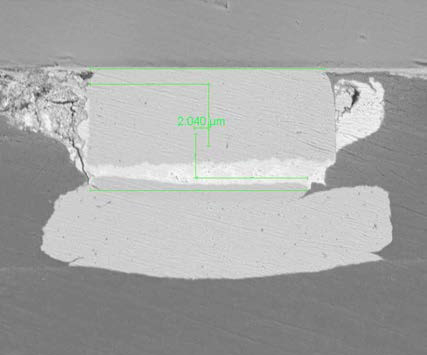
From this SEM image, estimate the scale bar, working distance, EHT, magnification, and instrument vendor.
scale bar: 30000 nm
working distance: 13.1 mm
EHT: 10 kV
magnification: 5 K X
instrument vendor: FEI